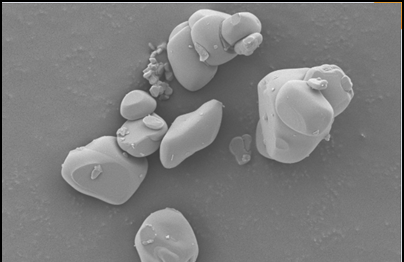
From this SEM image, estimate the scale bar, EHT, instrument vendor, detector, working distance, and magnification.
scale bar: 2000 nm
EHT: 3 kV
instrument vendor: Zeiss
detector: SE2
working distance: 7.9 mm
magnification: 5 K X